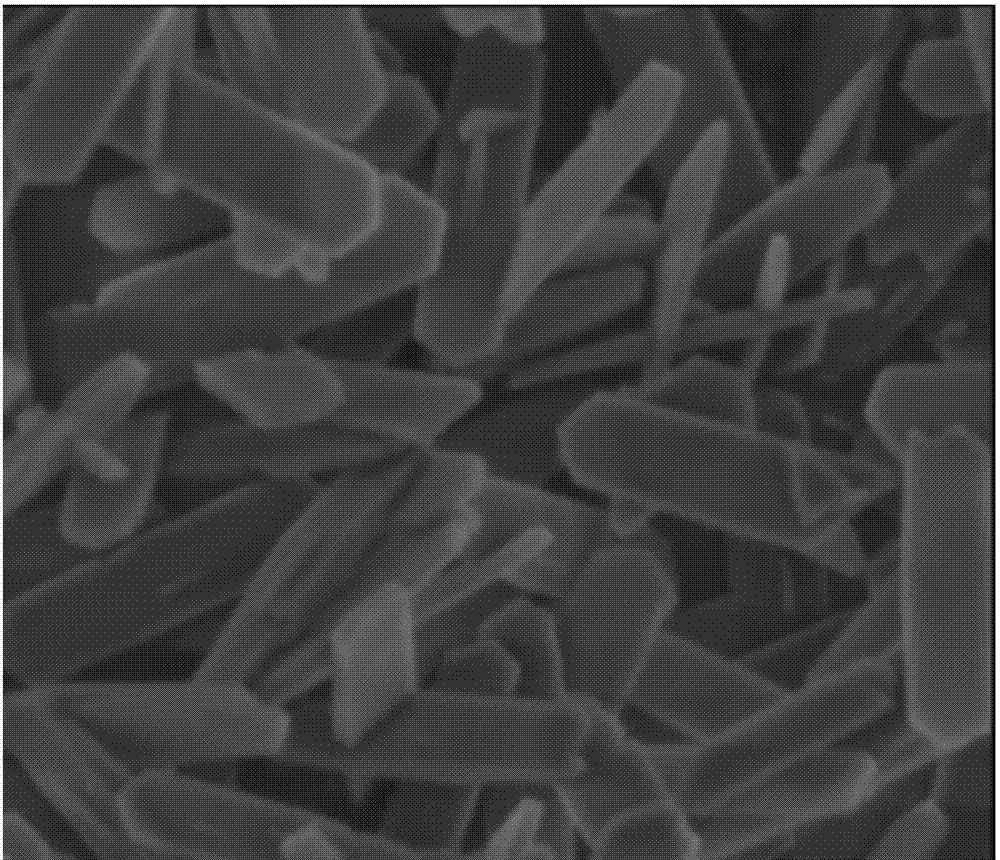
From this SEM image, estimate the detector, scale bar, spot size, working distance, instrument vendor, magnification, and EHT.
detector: ETD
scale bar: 2000 nm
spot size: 3.5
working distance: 4.6 mm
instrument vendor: FEI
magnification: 40 K X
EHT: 3 kV